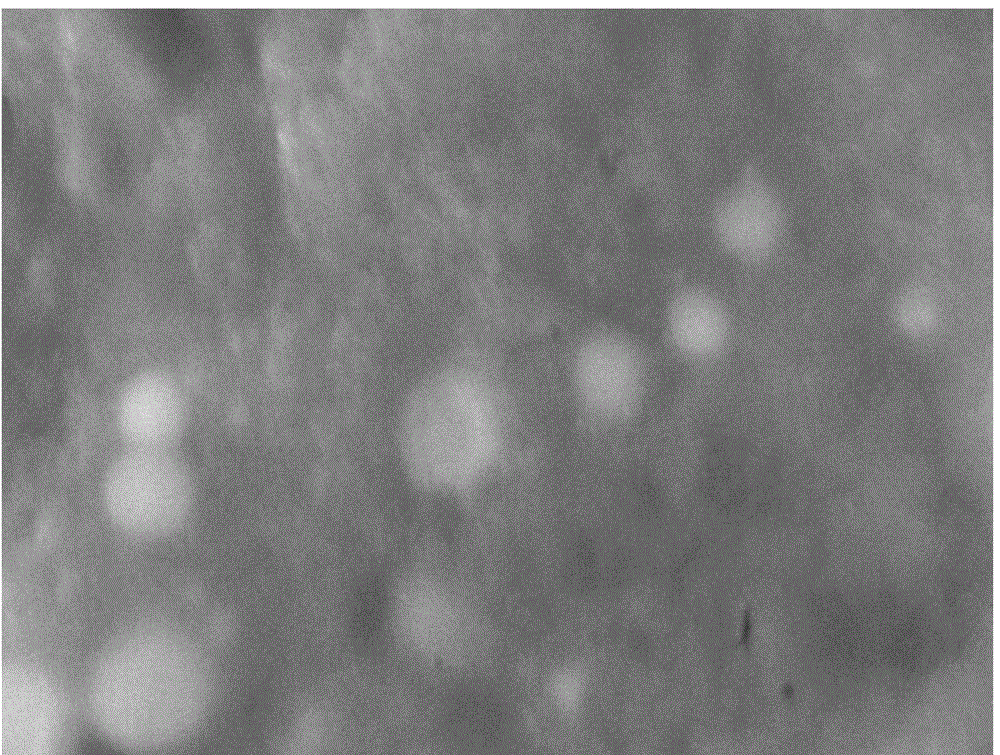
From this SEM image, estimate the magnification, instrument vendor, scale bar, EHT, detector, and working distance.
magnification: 60 K X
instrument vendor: JEOL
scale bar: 100 nm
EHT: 5 kV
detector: SEI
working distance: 8.3 mm